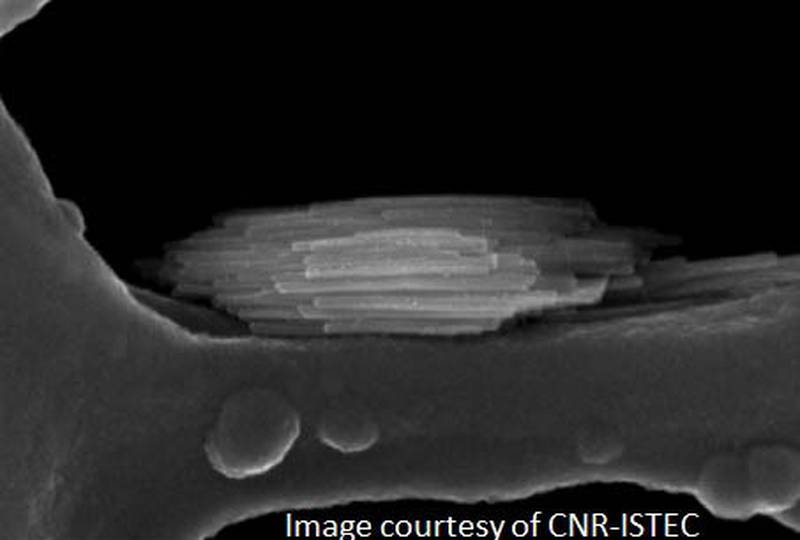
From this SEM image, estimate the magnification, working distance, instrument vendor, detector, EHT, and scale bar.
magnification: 500 K X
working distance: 1.8 mm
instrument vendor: Zeiss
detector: InLens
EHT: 10 kV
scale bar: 100 nm